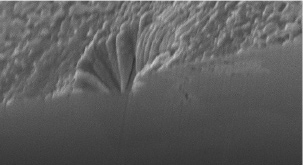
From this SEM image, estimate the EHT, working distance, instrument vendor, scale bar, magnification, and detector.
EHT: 10 kV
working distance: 10.5 mm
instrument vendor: Hitachi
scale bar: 5000 nm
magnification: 10 K X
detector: SE